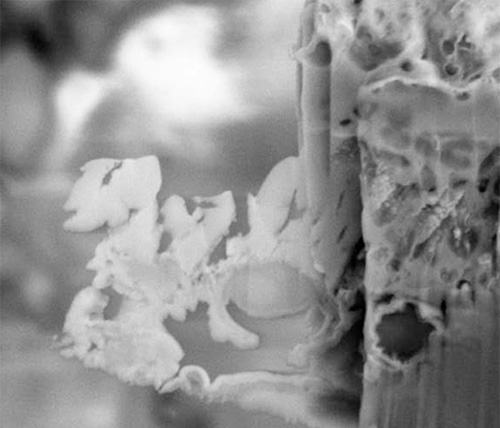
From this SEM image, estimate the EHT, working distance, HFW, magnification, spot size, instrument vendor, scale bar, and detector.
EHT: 10 kV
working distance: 5.116 mm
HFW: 15.2 µm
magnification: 20 K X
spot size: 4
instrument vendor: FEI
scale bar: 2000 nm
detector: SED